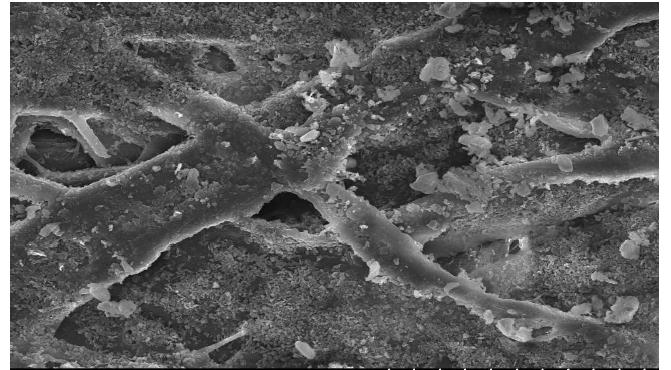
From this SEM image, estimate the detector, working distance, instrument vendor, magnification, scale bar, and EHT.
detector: SE(U)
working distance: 12 mm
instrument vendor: Hitachi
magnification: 1 K X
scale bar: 50000 nm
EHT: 15 kV